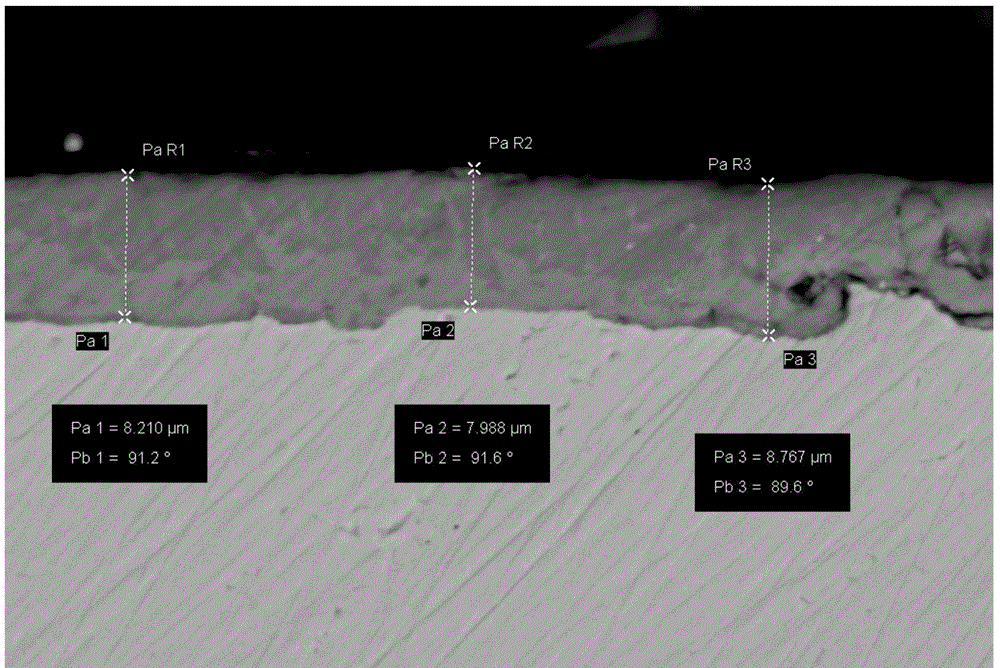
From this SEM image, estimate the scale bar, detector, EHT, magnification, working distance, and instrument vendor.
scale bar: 3000 nm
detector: CZ BSD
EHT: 20 kV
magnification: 2 K X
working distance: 10 mm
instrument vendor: Zeiss